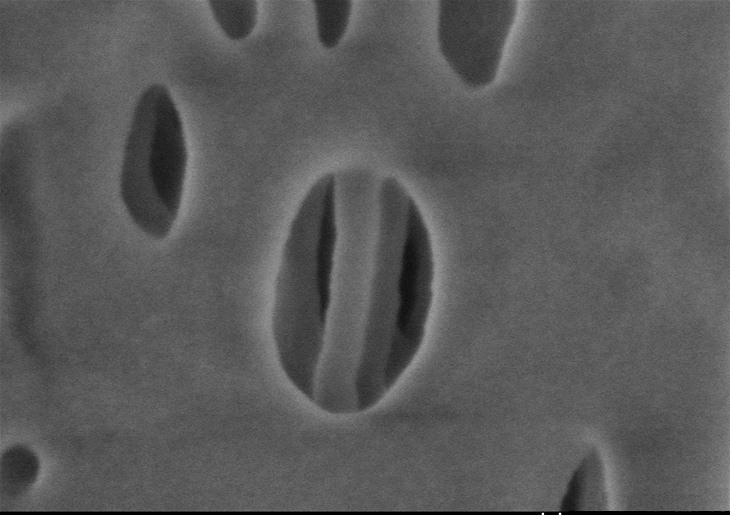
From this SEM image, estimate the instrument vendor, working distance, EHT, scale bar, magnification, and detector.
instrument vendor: Hitachi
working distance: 1.5 mm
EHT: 0.6 kV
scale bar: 100 nm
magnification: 300 K X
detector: SE(U,LA0)